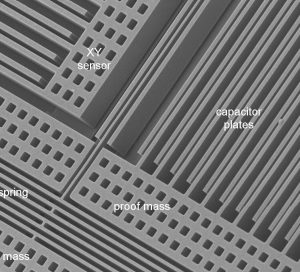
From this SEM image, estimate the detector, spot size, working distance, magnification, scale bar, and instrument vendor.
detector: SE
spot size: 3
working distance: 12.8 mm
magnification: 0.65 K X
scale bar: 20000 nm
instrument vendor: FEI